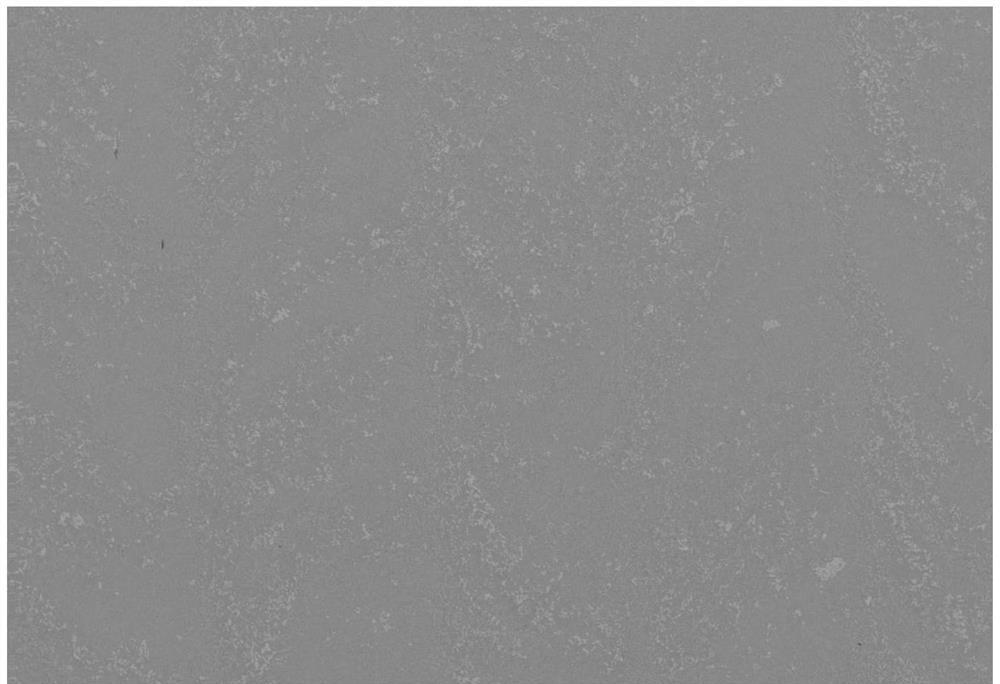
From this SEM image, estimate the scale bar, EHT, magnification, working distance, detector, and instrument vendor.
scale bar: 100000 nm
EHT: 20 kV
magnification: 0.1 K X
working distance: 9.1 mm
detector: SE2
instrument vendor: Zeiss